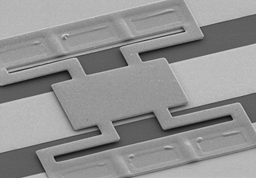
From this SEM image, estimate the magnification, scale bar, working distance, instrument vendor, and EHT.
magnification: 0.3 K X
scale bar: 100000 nm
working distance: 38.4 mm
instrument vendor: Hitachi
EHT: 10 kV